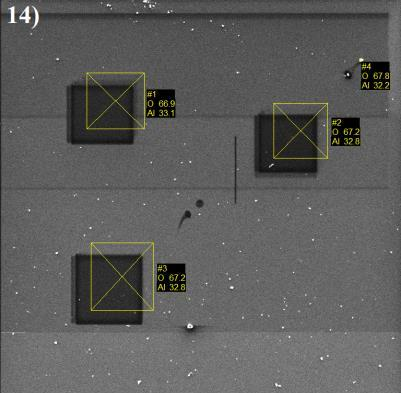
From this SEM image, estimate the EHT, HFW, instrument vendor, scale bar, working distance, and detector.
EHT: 5 kV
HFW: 171 µm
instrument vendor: Tescan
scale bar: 50000 nm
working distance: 15.3 mm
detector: SE